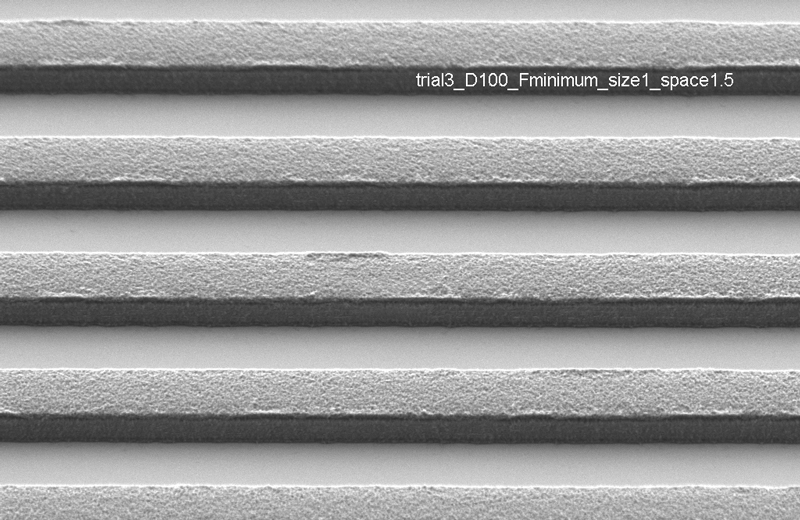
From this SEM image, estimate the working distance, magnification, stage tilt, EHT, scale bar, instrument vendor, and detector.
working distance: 6.6 mm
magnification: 9.66 K X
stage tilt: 45.4°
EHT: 1 kV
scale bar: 1000 nm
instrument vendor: Zeiss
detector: HE-SE2